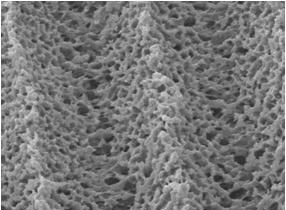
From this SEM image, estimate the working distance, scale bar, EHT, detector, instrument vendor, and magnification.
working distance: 8 mm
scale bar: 10000 nm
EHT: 10 kV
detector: LEI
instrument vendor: JEOL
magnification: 2.5 K X